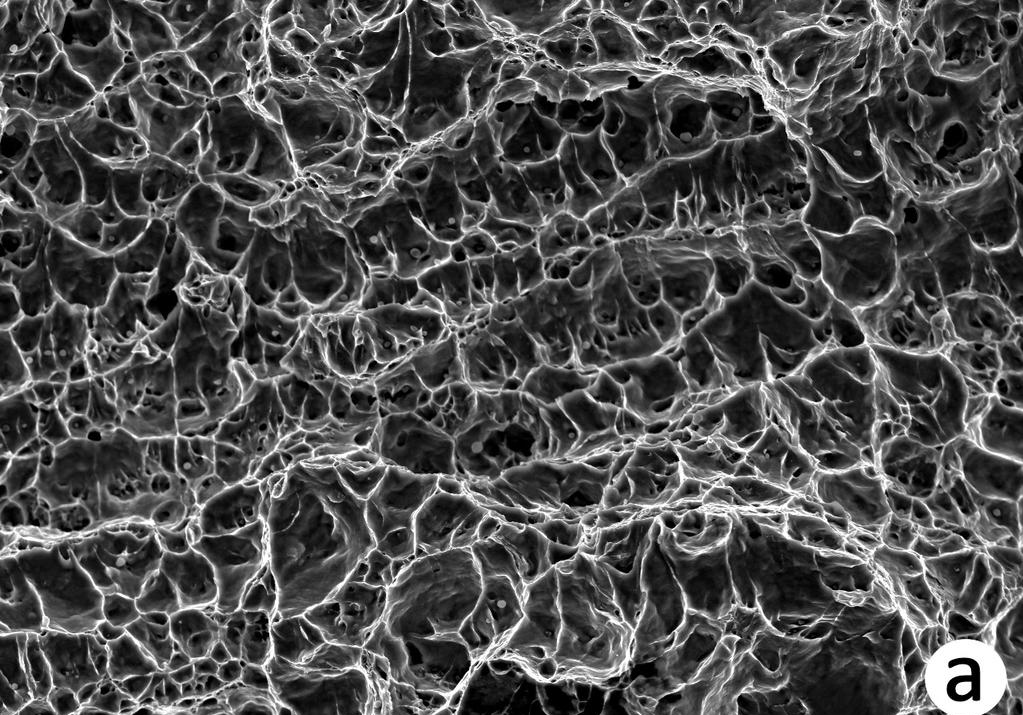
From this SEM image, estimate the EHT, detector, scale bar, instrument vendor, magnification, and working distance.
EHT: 15 kV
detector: LED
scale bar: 10000 nm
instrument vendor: JEOL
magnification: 1.5 K X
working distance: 10 mm